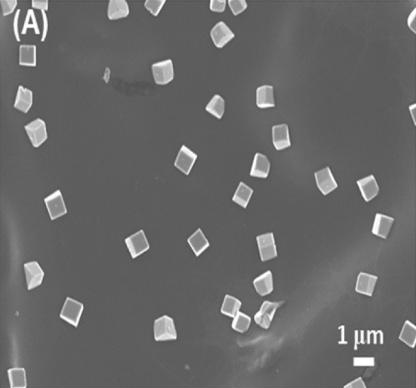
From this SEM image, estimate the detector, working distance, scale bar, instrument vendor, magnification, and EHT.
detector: SEI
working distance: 6.6 mm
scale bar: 1000 nm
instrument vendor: JEOL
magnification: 6 K X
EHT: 5 kV